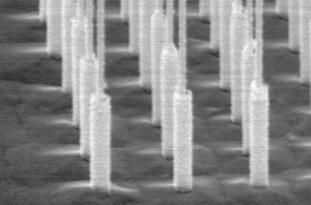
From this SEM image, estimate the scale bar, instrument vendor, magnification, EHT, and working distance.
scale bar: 1000 nm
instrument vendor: JEOL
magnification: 18 K X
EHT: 15 kV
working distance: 37 mm